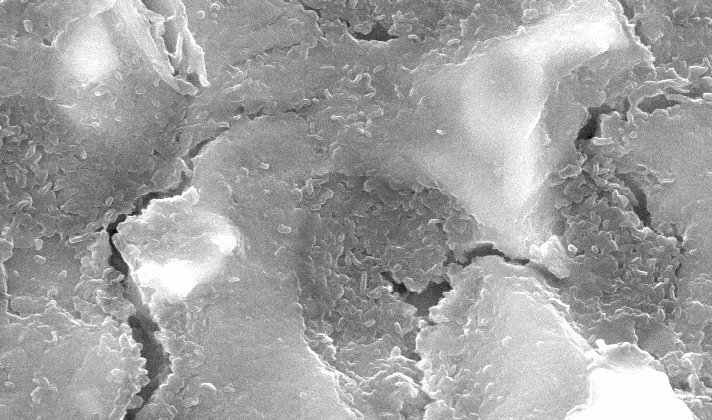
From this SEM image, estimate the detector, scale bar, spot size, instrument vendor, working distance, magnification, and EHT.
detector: SE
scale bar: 2000 nm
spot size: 2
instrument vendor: FEI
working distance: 10.2 mm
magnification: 6 K X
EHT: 20 kV